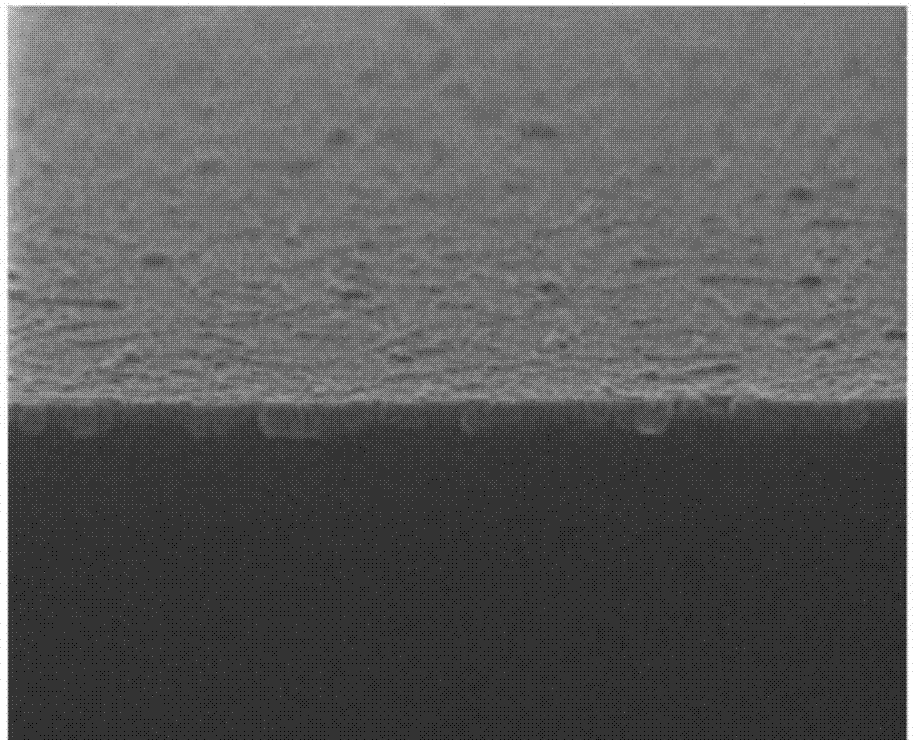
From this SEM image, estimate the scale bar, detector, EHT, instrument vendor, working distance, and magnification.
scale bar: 500 nm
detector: SE(U)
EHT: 5 kV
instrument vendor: Hitachi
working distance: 12 mm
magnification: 100 K X